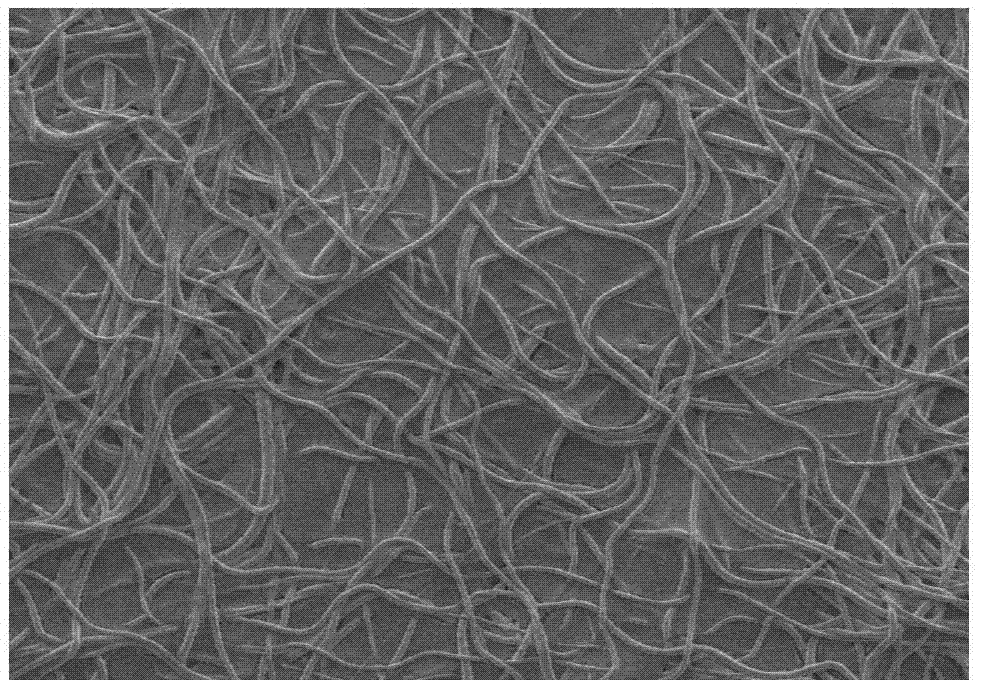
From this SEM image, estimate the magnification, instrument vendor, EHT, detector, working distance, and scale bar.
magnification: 3 K X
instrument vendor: JEOL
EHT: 1 kV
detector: LED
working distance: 7.8 mm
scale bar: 1000 nm